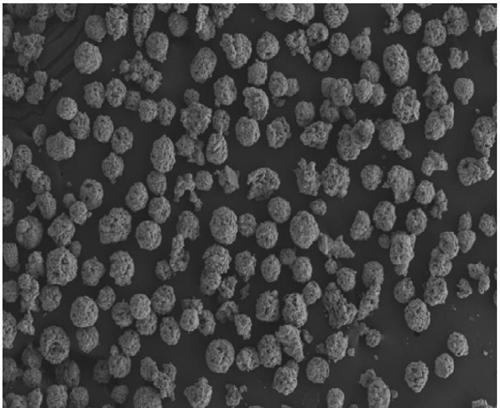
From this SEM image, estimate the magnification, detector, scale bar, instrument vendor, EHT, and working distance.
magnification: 0.15 K X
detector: SE2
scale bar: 100000 nm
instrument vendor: Zeiss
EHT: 15 kV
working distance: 9.5 mm